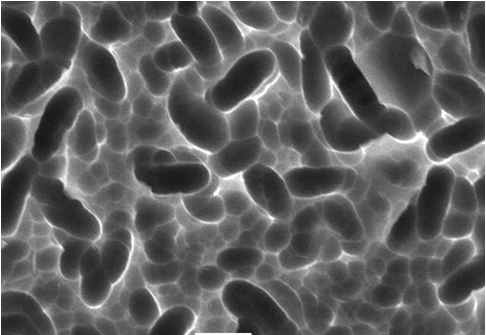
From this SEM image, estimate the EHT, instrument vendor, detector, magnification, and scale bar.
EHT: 20 kV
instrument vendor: JEOL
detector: SEI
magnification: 3 K X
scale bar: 5000 nm